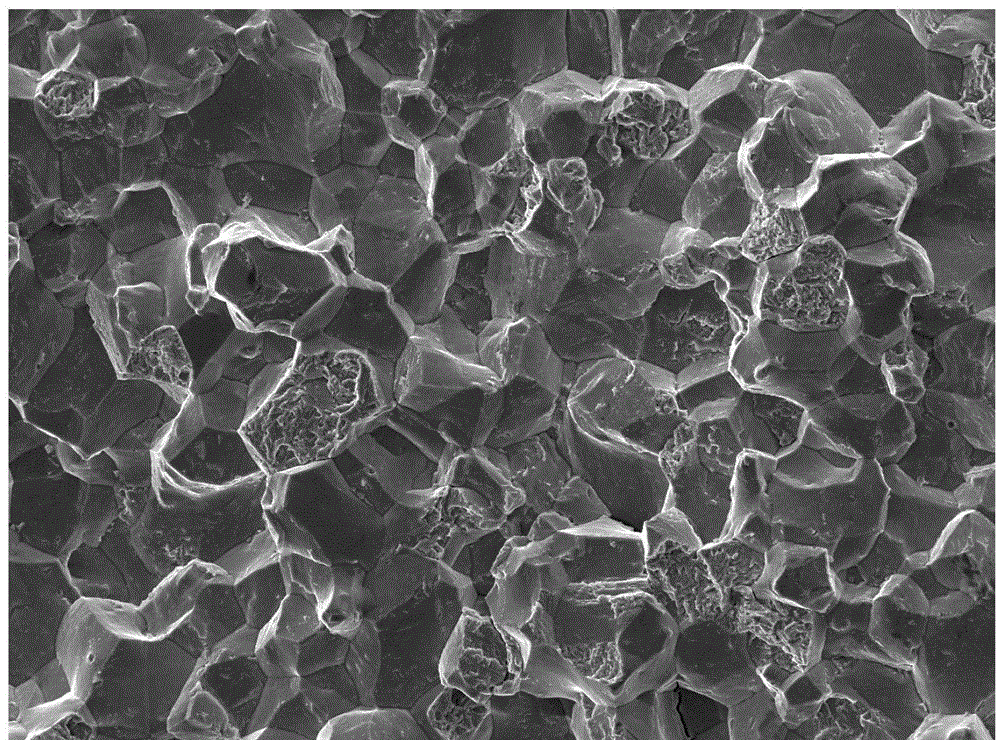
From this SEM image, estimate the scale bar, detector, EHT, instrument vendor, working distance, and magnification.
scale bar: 10000 nm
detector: SEI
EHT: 20 kV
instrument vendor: JEOL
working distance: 9 mm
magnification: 0.5 K X